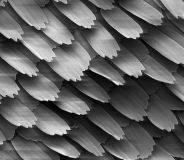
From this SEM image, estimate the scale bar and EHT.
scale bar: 200000 nm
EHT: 5 kV